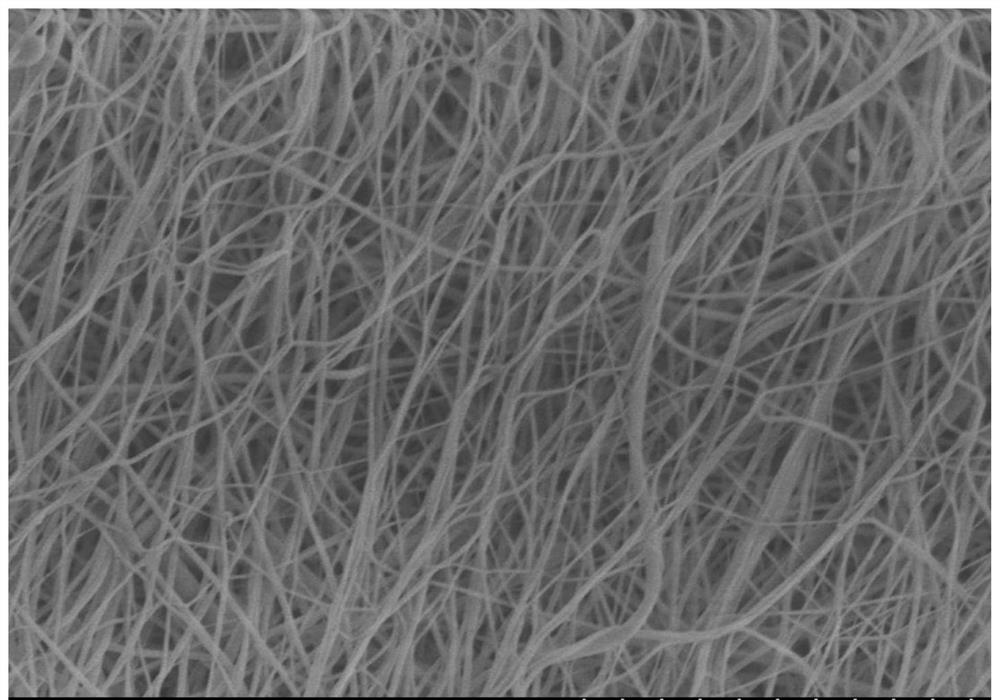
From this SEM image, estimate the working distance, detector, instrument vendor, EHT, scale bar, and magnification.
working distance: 13 mm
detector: SE(M)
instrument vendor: Hitachi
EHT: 3 kV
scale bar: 5000 nm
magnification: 10 K X